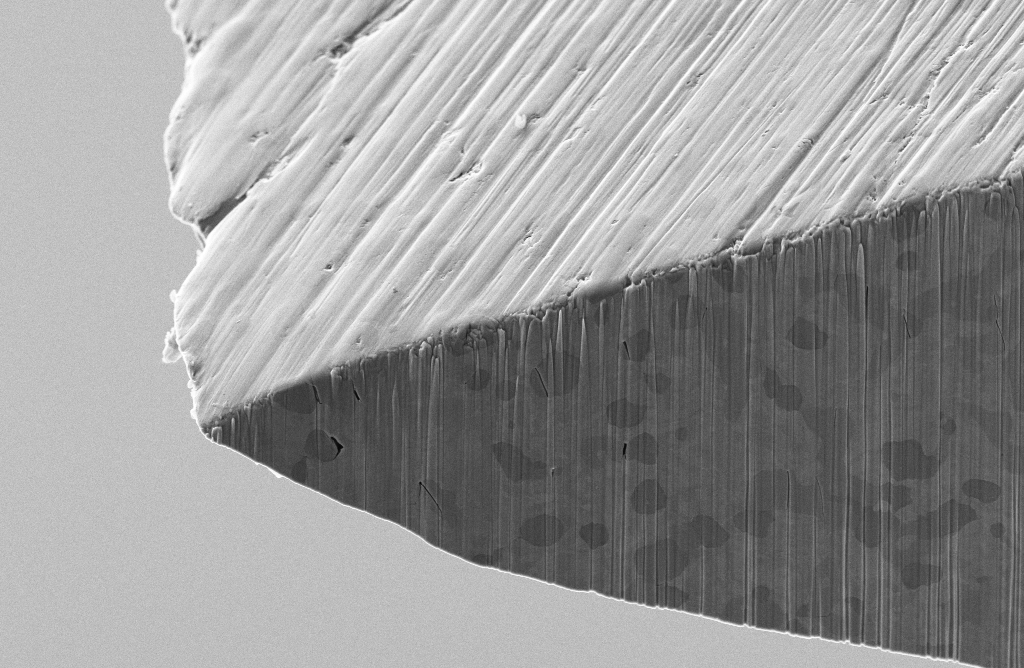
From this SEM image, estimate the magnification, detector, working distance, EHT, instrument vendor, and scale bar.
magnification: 3 K X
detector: SE2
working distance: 6.6 mm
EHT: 5 kV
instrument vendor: Zeiss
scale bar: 2000 nm